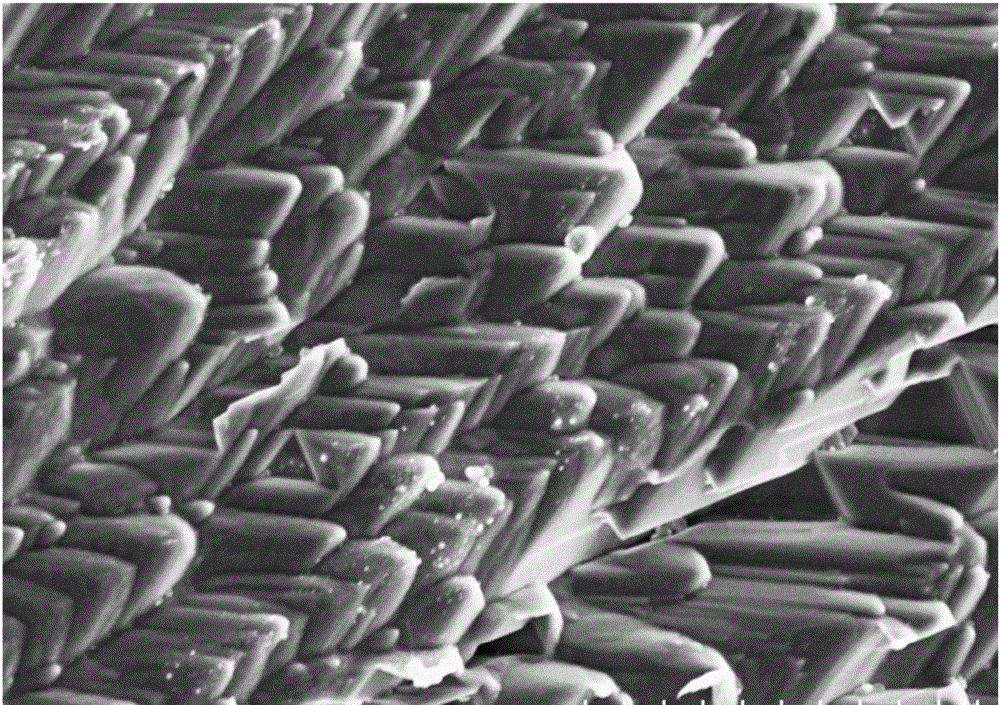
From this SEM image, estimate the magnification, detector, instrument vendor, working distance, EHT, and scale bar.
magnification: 5 K X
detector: SE(M)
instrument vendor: Hitachi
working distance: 12.4 mm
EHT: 5 kV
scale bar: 10000 nm